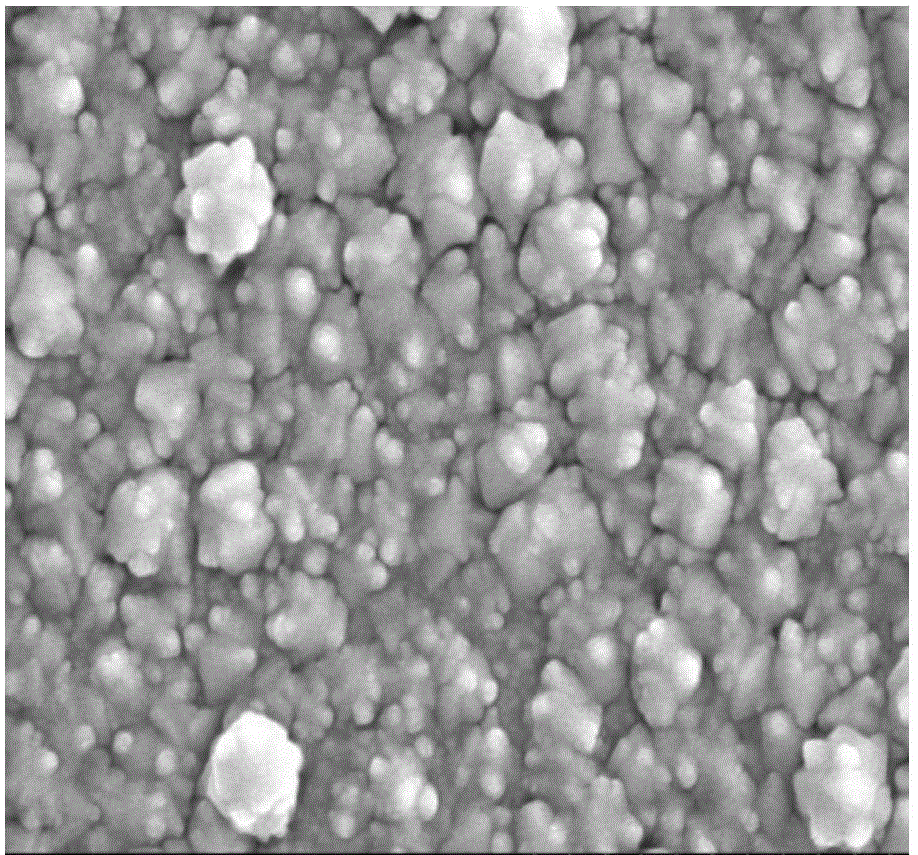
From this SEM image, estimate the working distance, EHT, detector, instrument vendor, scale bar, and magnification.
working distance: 9.9 mm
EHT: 5 kV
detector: SE(M)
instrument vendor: Hitachi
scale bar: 1000 nm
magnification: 50 K X